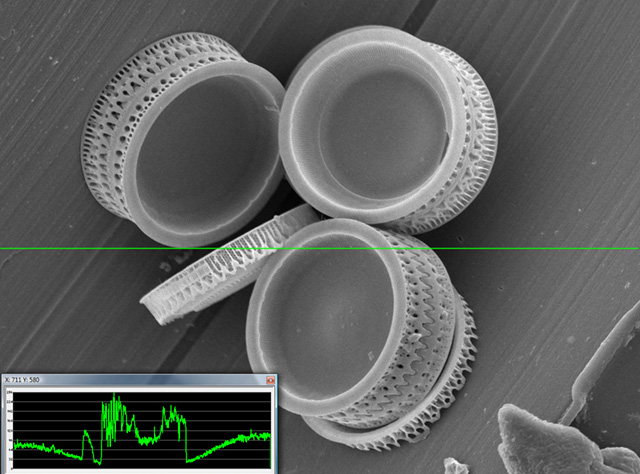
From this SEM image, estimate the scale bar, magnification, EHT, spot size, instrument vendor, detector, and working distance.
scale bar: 10000 nm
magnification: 1.8 K X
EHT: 10 kV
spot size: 7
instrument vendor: JEOL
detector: SEI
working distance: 20 mm